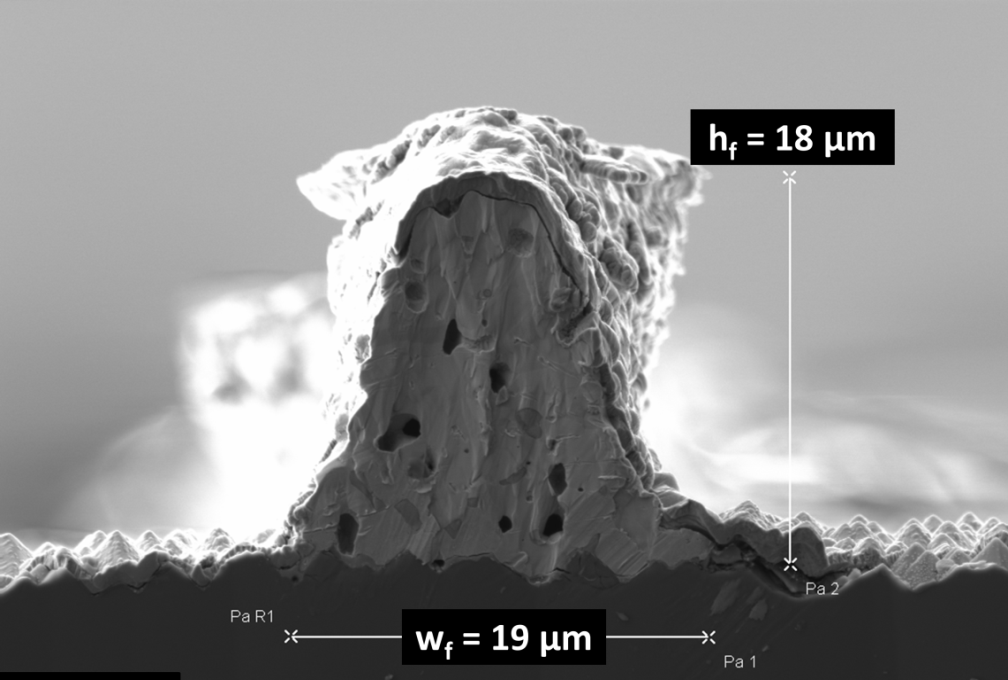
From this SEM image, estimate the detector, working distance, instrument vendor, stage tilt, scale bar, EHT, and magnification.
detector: SE2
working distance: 4.7 mm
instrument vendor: Zeiss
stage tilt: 0°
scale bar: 5000 nm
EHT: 5 kV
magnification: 2.5 K X